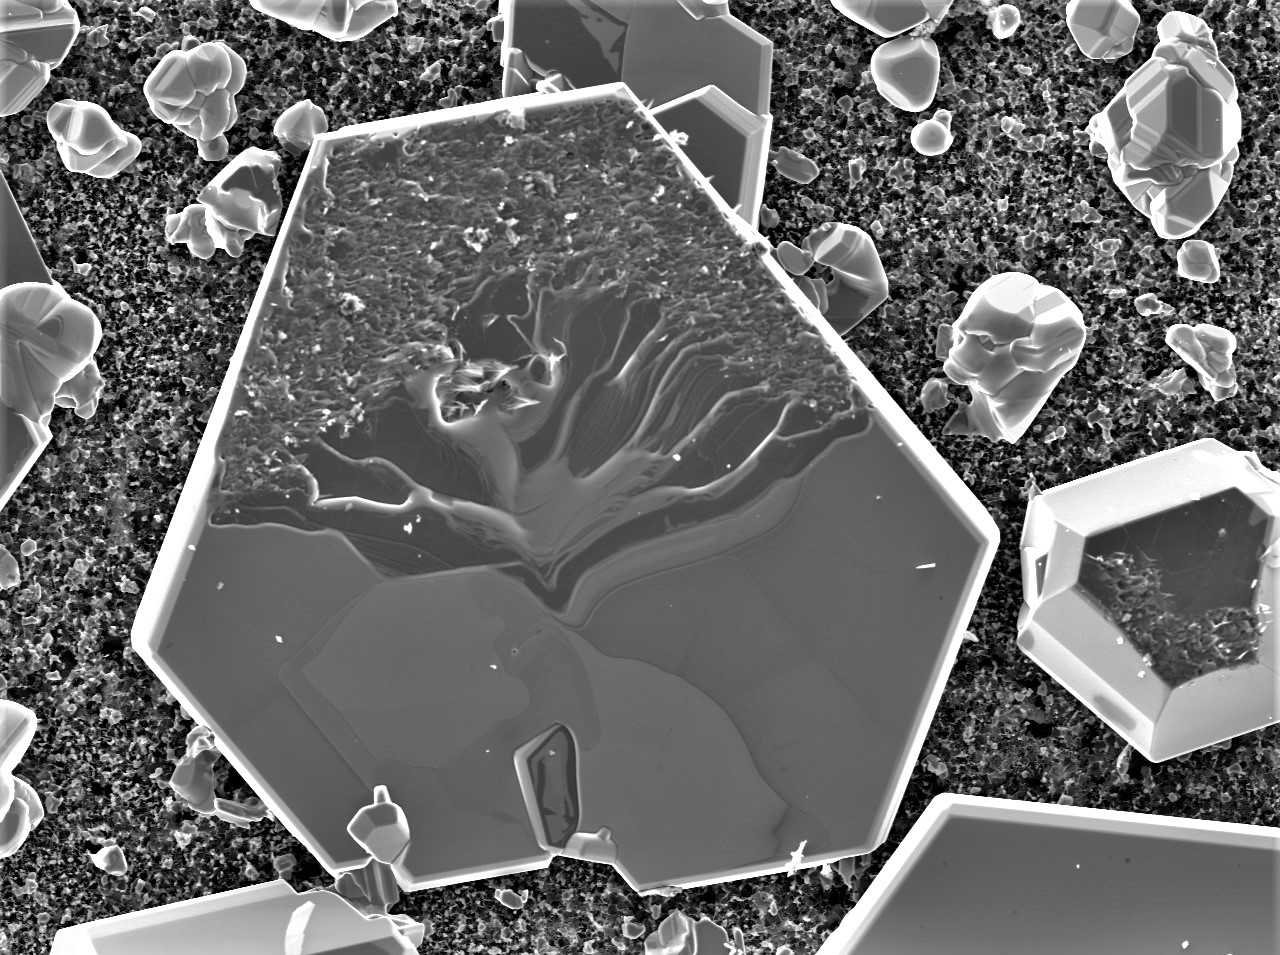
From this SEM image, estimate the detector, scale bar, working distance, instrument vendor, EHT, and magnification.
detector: SED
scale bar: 50000 nm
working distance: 9.1 mm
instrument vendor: JEOL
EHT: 10 kV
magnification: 0.43 K X